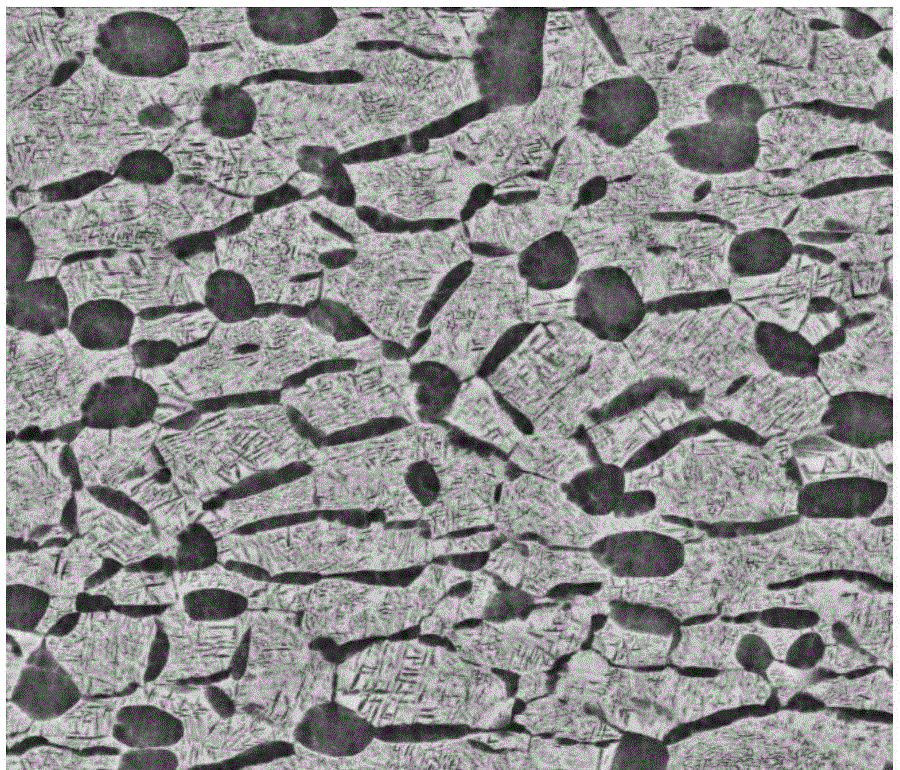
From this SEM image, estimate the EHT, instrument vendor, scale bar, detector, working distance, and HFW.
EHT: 20 kV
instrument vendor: FEI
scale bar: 10000 nm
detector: BSED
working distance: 10.9 mm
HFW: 29.8 µm